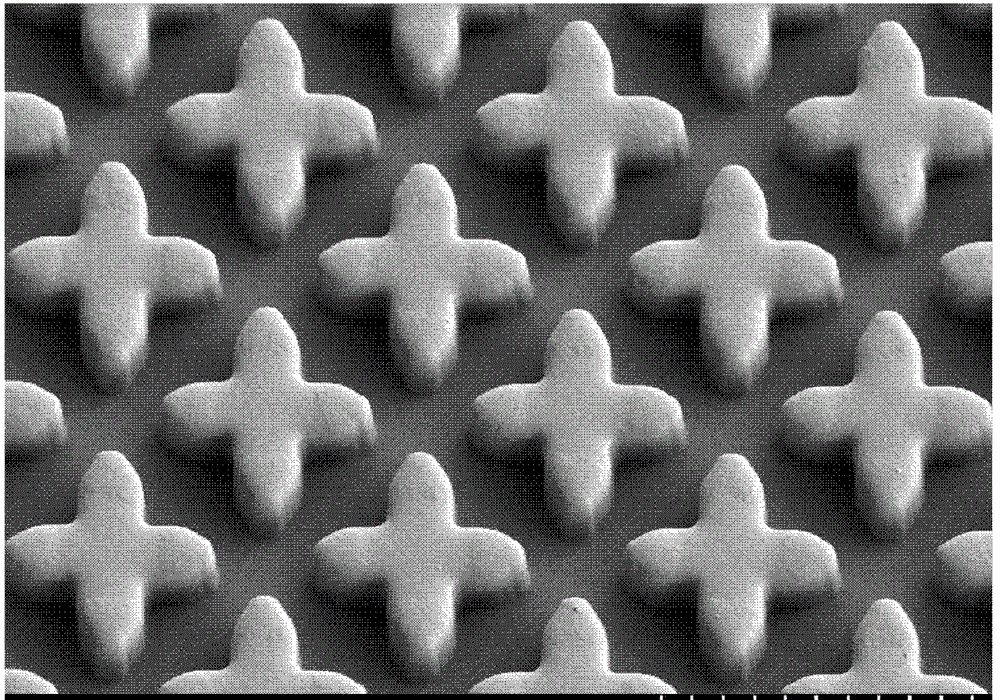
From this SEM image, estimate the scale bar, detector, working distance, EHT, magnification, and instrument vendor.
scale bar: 20000 nm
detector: SE(L)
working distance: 9.4 mm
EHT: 1 kV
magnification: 2 K X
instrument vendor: Hitachi